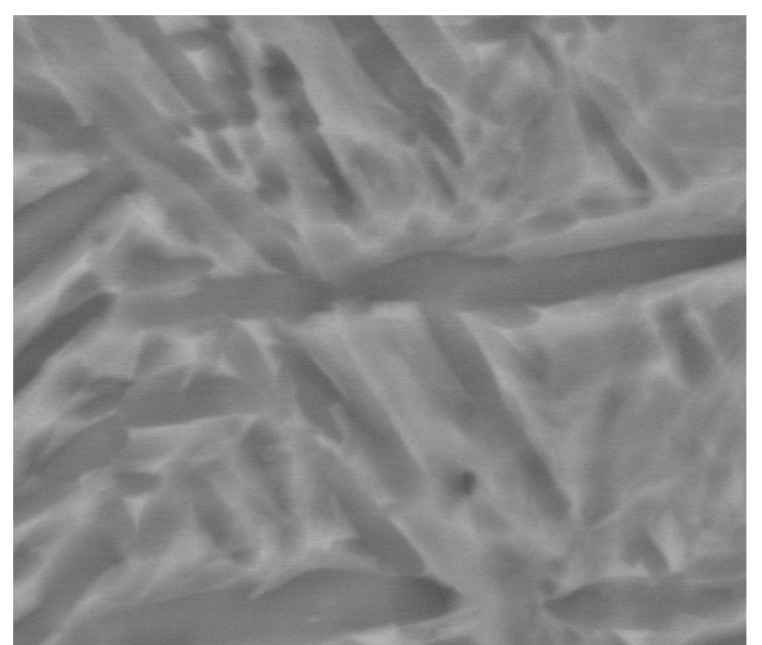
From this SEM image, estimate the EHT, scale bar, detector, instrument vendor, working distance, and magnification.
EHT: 20 kV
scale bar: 5000 nm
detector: vCD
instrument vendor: FEI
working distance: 9.7 mm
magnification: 8.003 K X